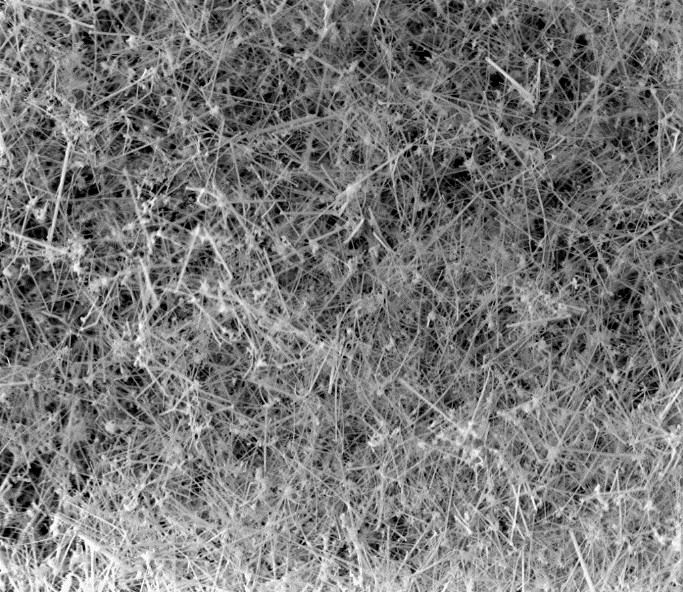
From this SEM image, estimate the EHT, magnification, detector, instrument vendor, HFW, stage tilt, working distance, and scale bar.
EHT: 5 kV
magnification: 10 K X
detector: TLD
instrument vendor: FEI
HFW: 12.7 µm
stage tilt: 0°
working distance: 4 mm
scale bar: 3000 nm